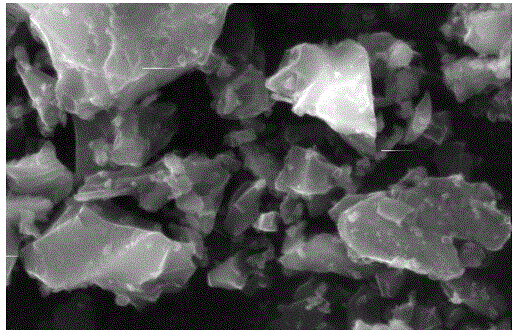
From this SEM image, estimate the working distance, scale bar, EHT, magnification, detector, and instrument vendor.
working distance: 8 mm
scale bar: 5000 nm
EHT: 20 kV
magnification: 10 K X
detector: SE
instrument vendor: FEI